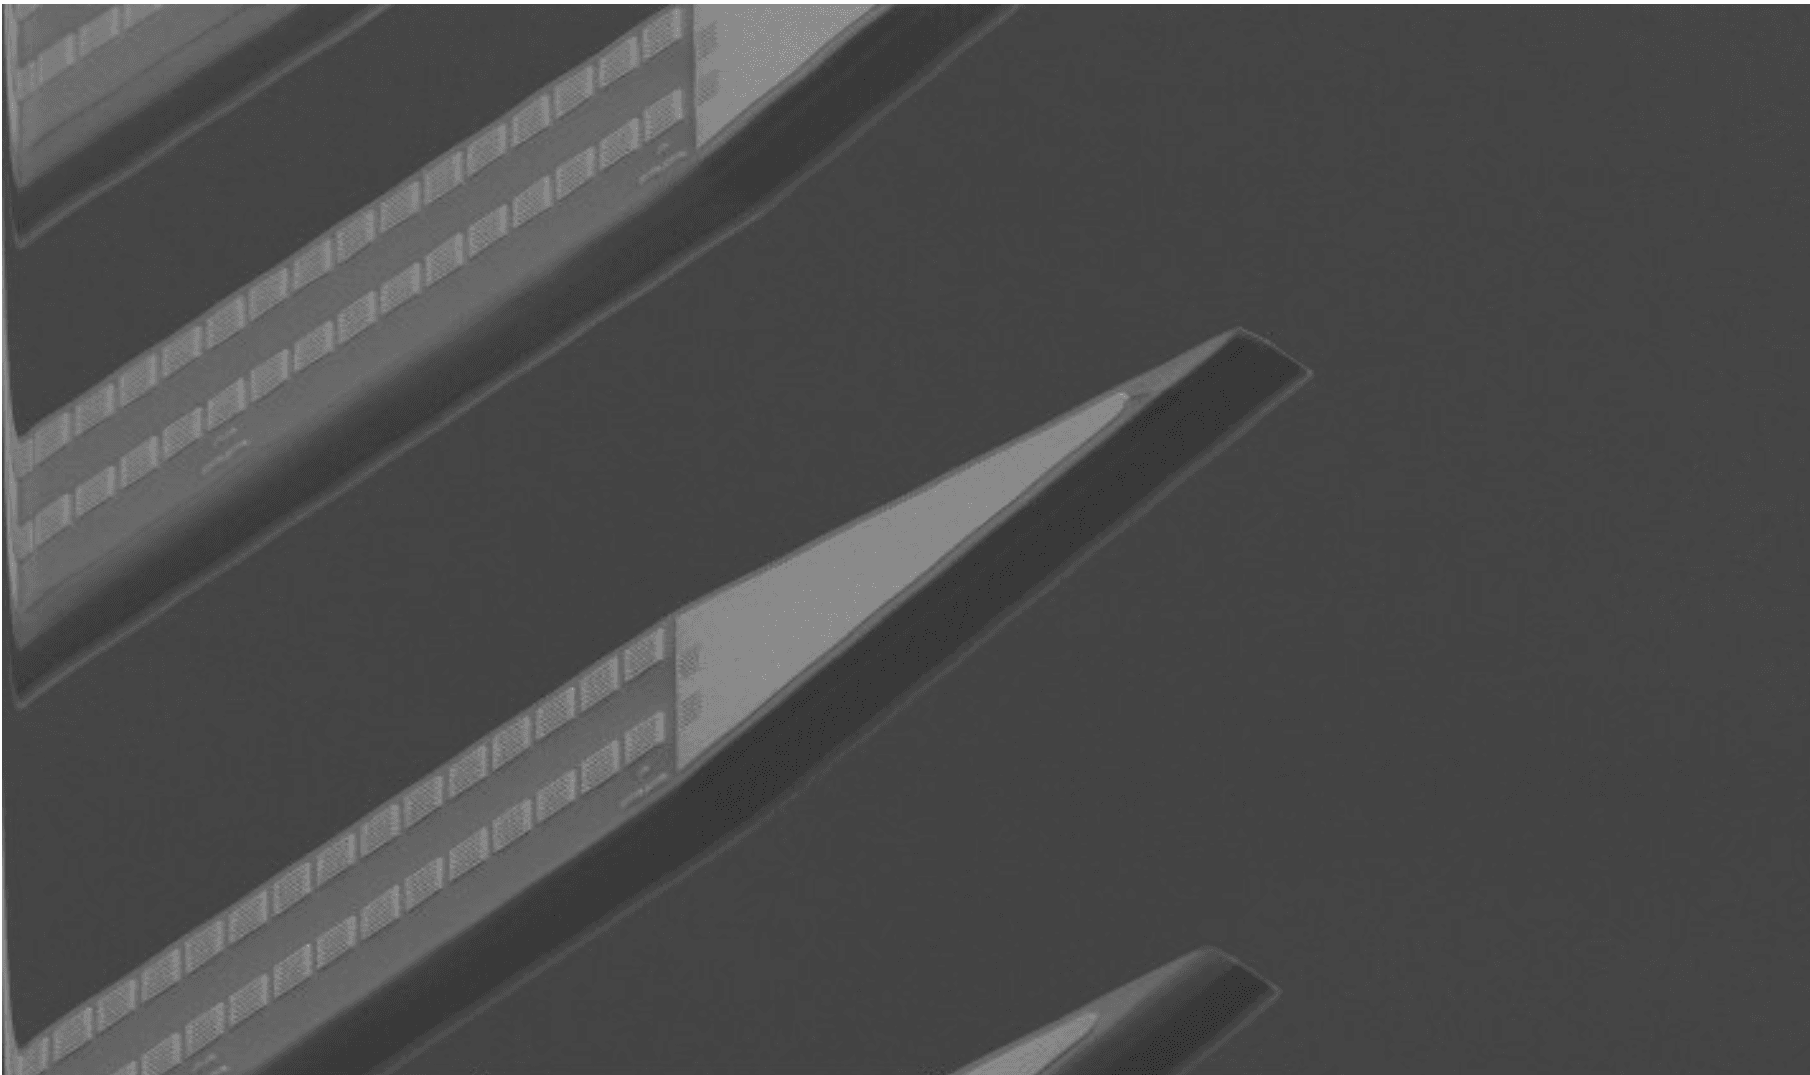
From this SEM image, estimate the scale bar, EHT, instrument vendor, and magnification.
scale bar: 100000 nm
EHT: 5 kV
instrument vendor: FEI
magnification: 0.6 K X